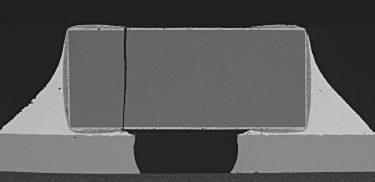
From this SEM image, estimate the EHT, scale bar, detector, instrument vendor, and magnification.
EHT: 20 kV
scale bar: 500000 nm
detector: BSE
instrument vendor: Tescan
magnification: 0.1 K X